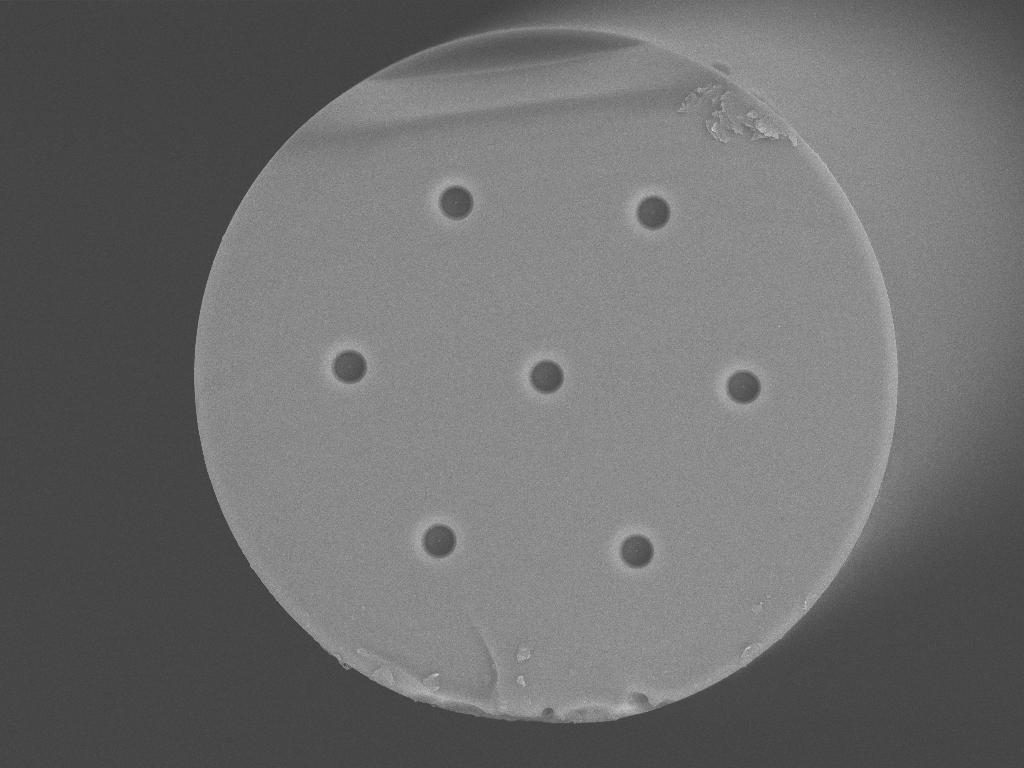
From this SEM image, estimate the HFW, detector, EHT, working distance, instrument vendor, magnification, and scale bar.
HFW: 182 µm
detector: BSE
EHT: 20 kV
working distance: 6.6 mm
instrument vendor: Tescan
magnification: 1.39 K X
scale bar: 50000 nm